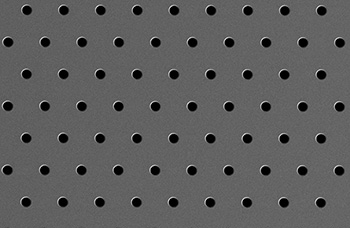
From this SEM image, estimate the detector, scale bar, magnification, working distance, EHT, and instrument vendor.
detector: SE2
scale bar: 10000 nm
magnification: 1 K X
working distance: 5.1 mm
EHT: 2 kV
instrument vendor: Zeiss